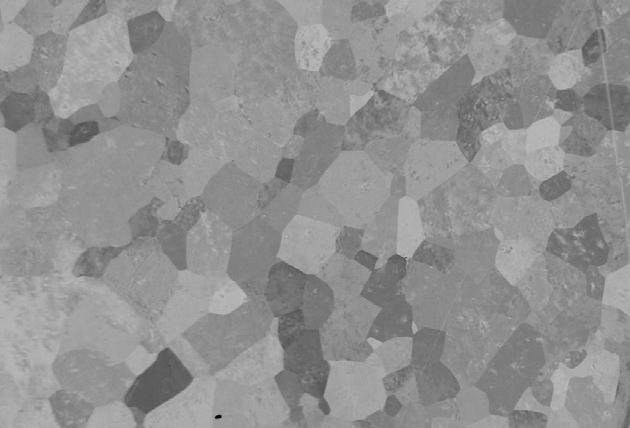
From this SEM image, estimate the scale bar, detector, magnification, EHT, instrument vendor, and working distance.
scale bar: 20000 nm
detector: QBSD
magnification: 0.5 K X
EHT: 20 kV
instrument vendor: Zeiss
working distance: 16 mm